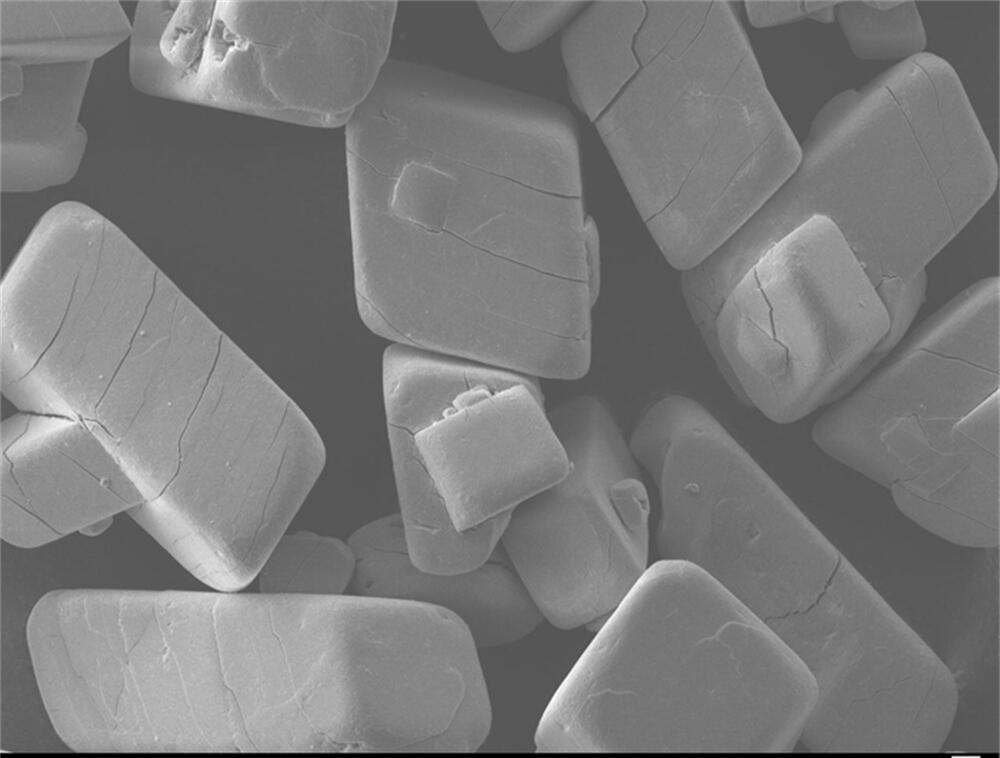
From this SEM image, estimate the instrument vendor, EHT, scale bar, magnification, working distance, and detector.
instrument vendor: JEOL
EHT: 10 kV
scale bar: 10000 nm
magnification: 0.35 K X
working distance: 8 mm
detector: LEI